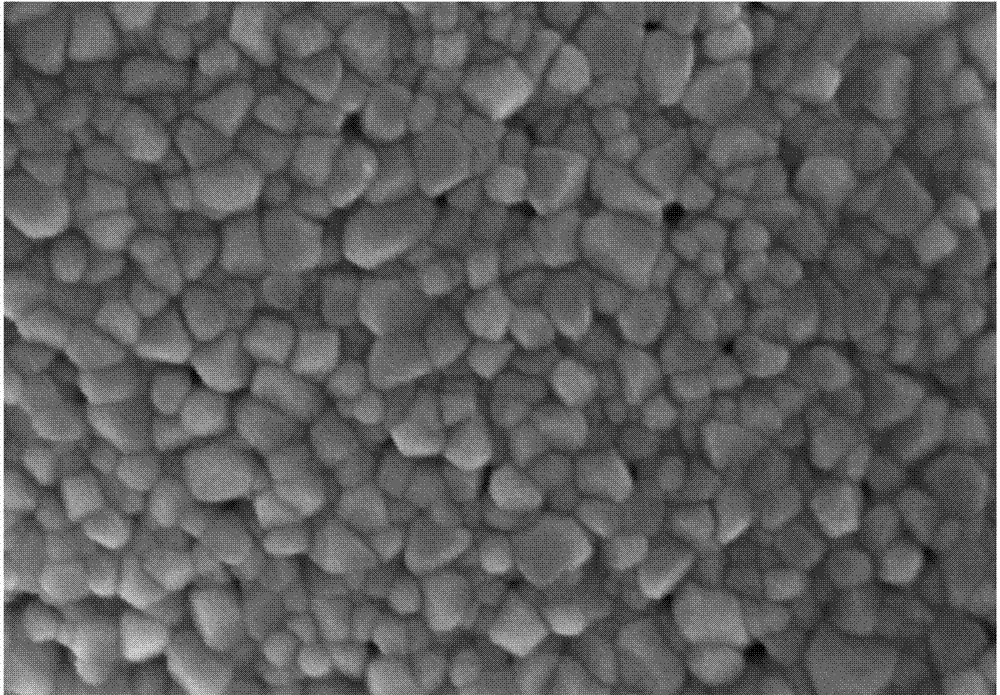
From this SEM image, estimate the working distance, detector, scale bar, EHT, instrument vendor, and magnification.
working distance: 8.2 mm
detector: SE(M)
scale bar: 500 nm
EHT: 7 kV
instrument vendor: Hitachi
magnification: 110 K X